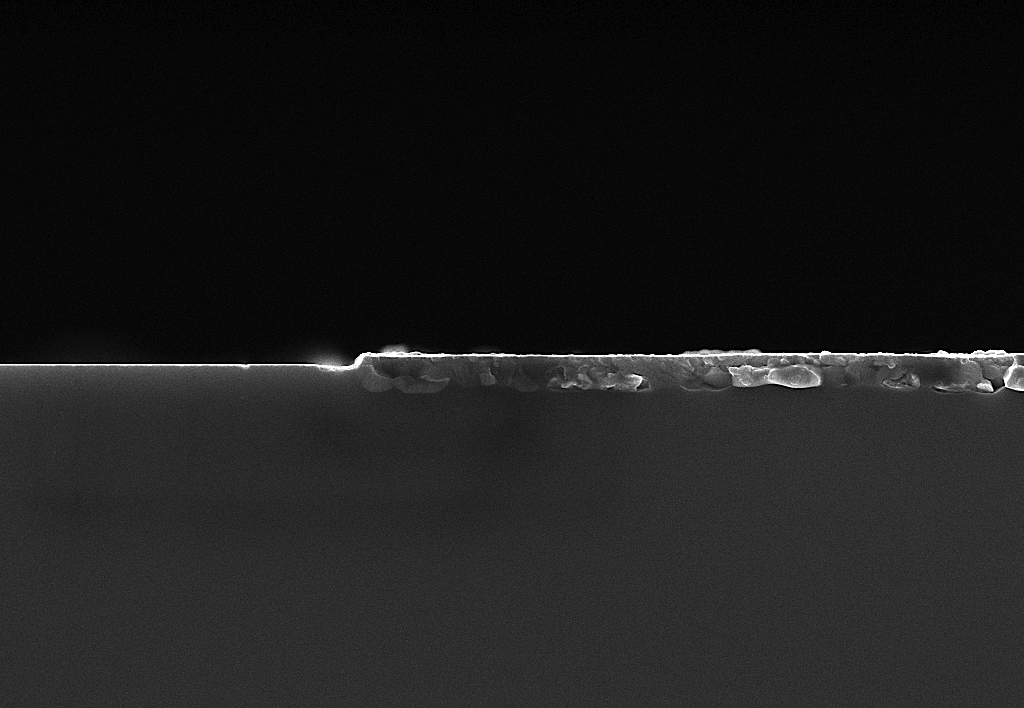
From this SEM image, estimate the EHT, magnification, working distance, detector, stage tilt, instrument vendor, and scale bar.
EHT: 7 kV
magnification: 50 K X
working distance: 1.9 mm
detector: InLens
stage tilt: -2.6°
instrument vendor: Zeiss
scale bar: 200 nm